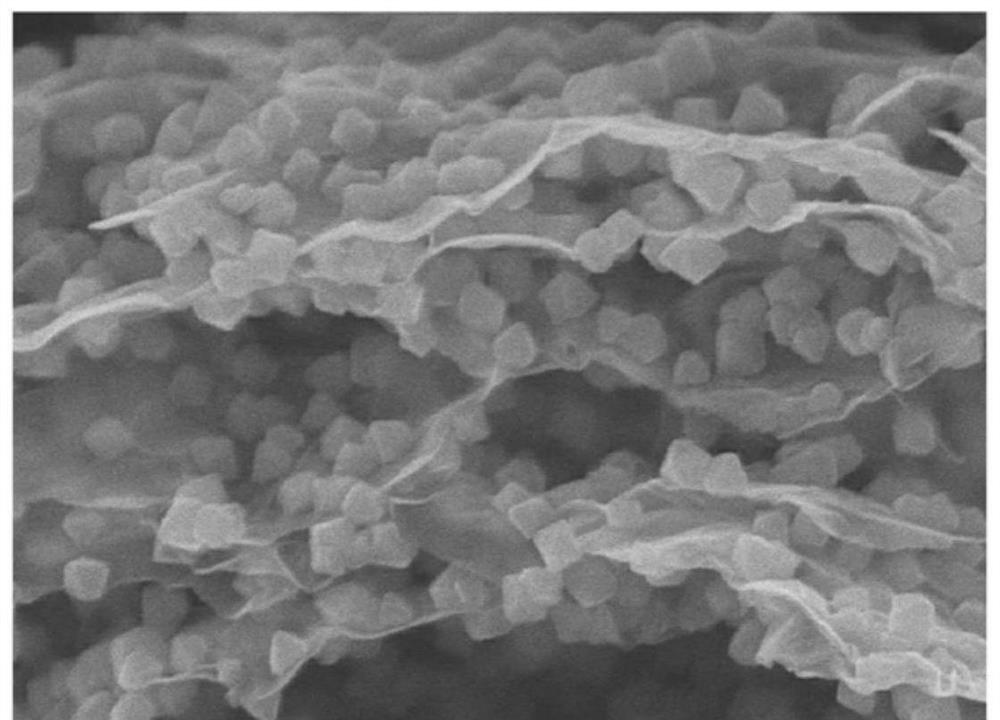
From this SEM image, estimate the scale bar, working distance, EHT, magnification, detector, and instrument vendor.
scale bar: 500 nm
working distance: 6.5 mm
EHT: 5 kV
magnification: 60 K X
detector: SE(UL)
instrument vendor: Hitachi